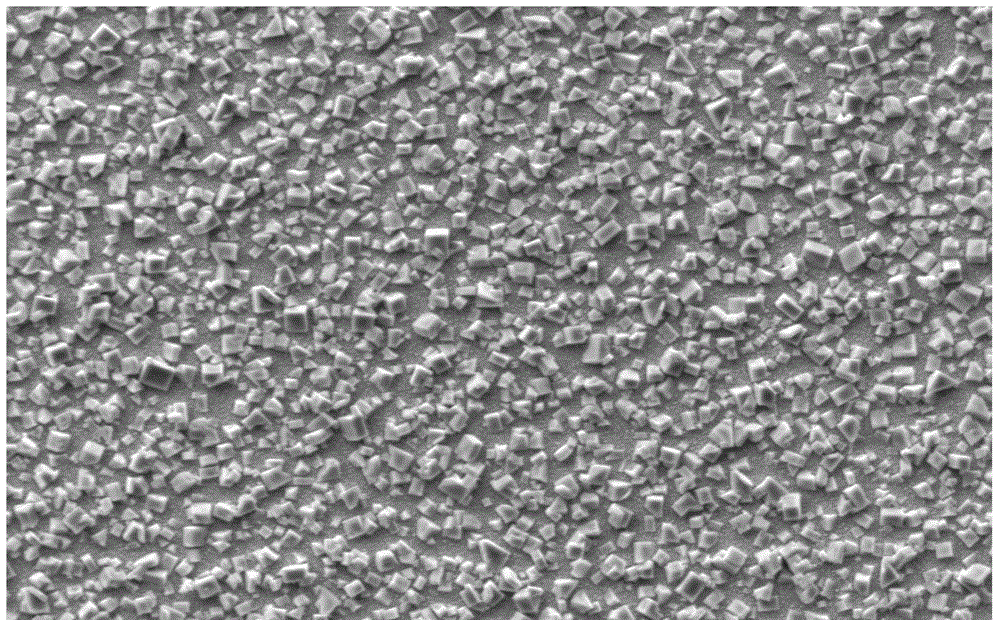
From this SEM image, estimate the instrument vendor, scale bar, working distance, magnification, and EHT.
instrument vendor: Zeiss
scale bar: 10000 nm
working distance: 12 mm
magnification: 2 K X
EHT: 15 kV